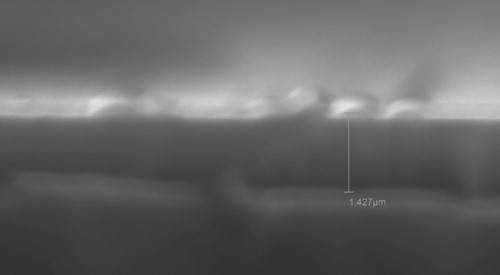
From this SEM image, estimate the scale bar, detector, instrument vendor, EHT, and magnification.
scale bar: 1000 nm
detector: SEI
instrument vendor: JEOL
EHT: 20 kV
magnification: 15 K X